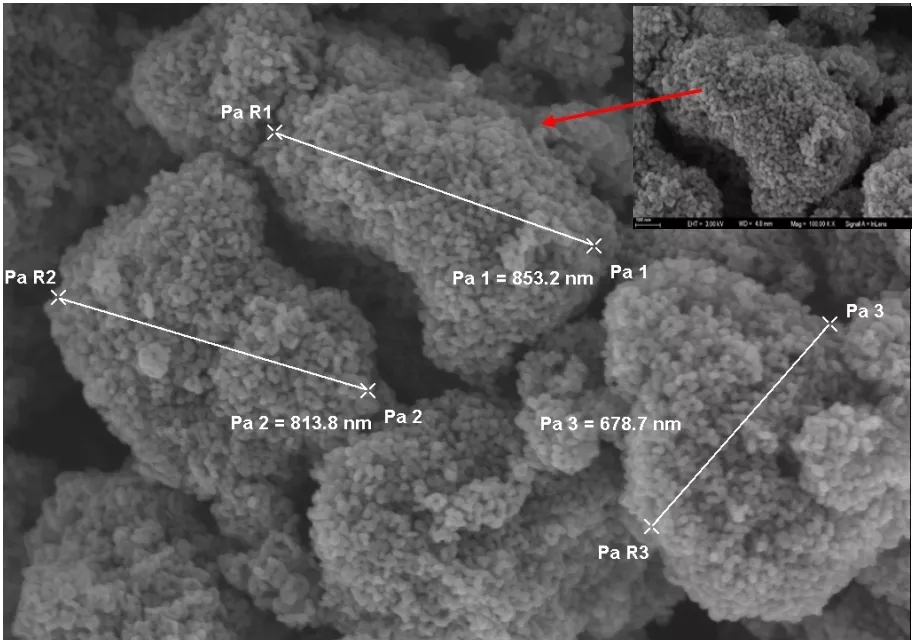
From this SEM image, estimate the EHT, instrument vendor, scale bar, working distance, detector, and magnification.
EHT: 3 kV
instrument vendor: Zeiss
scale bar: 100 nm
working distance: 4.8 mm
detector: InLens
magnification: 50 K X